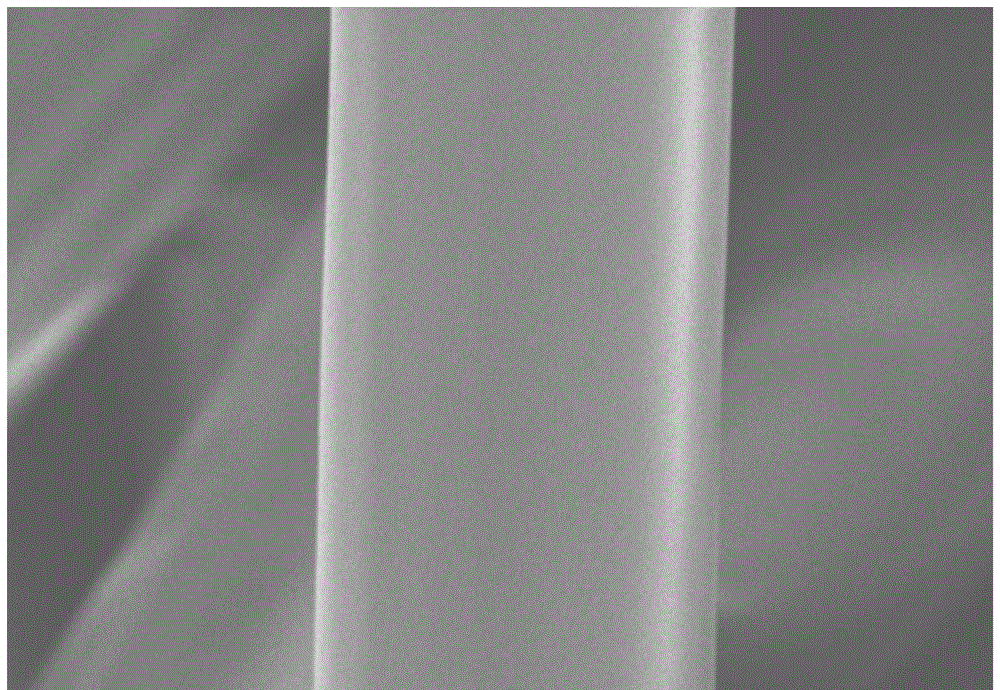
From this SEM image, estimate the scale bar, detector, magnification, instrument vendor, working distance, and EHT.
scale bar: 10000 nm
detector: SE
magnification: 4 K X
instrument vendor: Hitachi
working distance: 10.4 mm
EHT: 5 kV